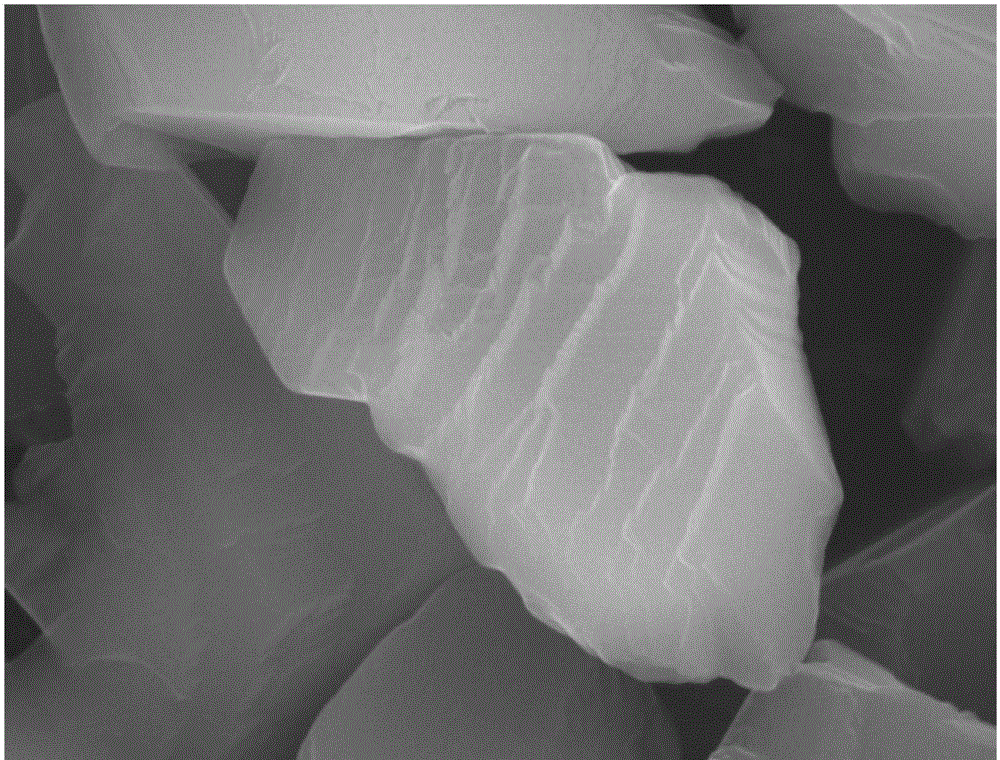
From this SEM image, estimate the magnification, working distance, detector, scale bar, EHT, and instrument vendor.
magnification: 20 K X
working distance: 8.6 mm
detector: SEI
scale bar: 1000 nm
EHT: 20 kV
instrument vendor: JEOL